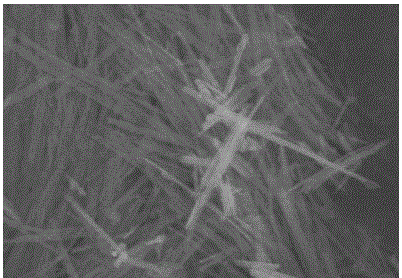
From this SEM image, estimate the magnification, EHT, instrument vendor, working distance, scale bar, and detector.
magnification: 50 K X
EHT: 5 kV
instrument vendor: Hitachi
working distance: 9.5 mm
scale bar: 1000 nm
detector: SE(U)